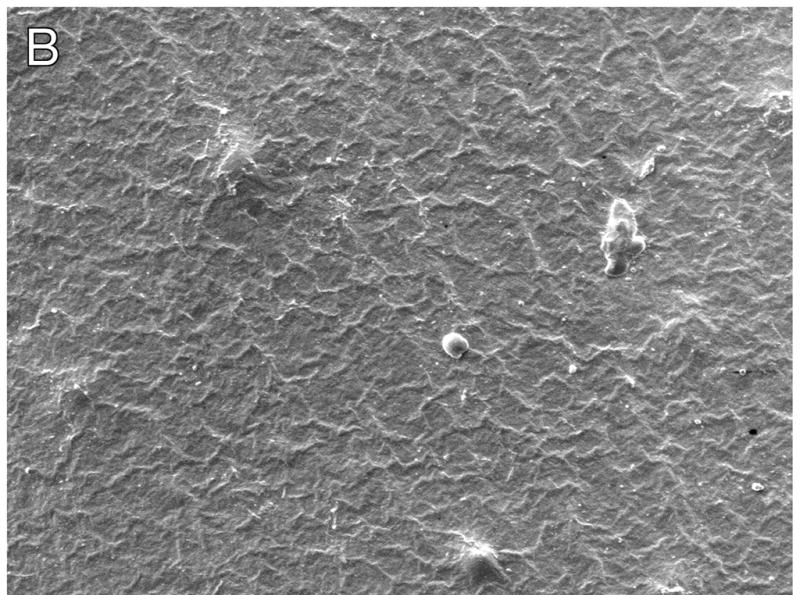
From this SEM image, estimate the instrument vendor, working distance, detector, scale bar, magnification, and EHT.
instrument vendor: JEOL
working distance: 7.7 mm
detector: LEI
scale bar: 10000 nm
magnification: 0.5 K X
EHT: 5 kV